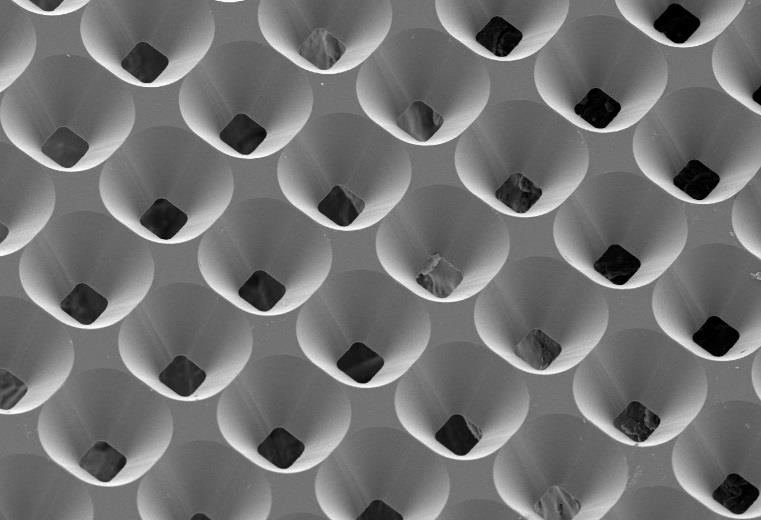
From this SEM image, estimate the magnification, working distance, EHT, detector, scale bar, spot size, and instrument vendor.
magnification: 0.27 K X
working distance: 34 mm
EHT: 10 kV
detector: SEI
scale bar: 50000 nm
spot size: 50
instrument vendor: JEOL